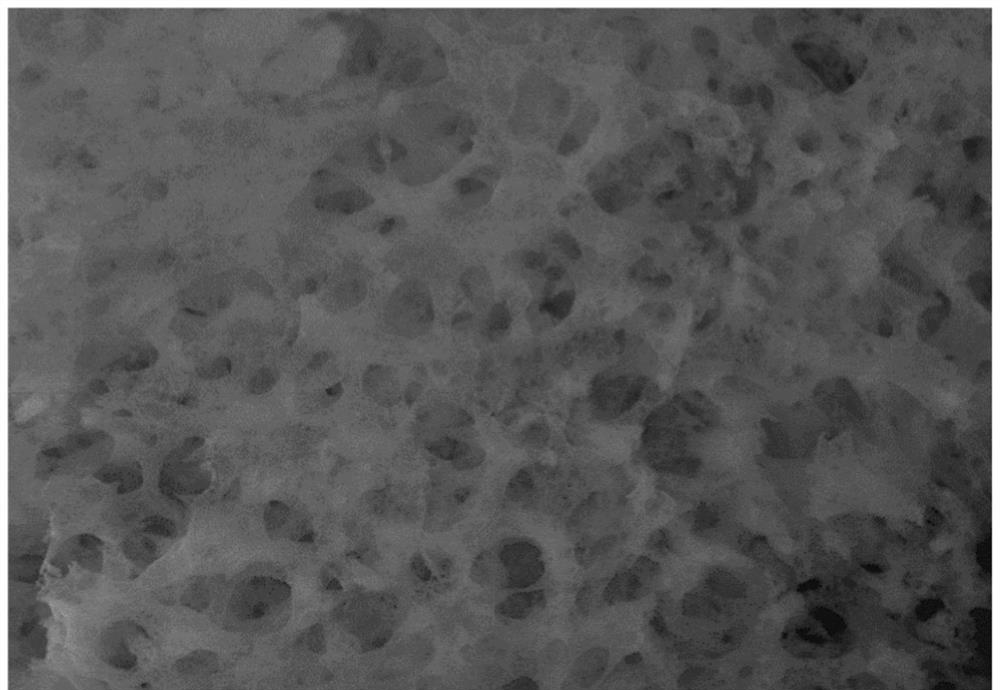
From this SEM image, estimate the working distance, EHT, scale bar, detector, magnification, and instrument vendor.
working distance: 10.7 mm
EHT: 20 kV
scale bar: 1000 nm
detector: InLens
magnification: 5 K X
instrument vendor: Zeiss